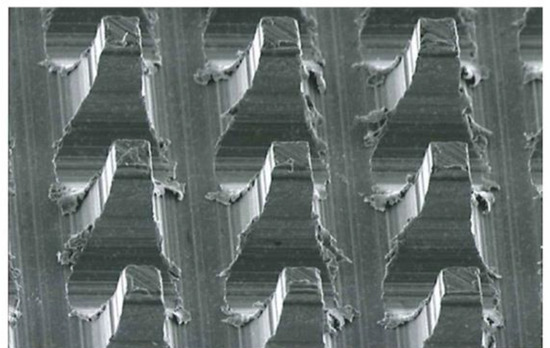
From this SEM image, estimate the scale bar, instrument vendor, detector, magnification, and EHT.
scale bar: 50000 nm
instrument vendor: JEOL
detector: SEI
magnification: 0.5 K X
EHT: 10 kV